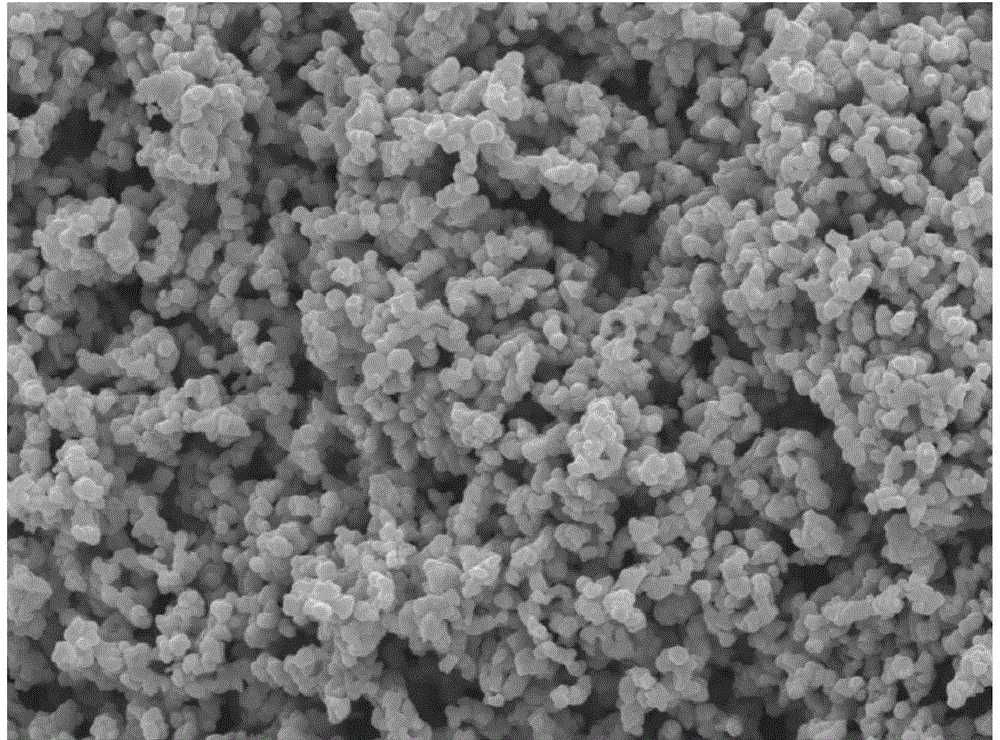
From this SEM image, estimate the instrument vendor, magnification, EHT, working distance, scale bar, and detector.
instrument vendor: JEOL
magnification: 10 K X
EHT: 10 kV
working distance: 9.7 mm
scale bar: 1000 nm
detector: SEI